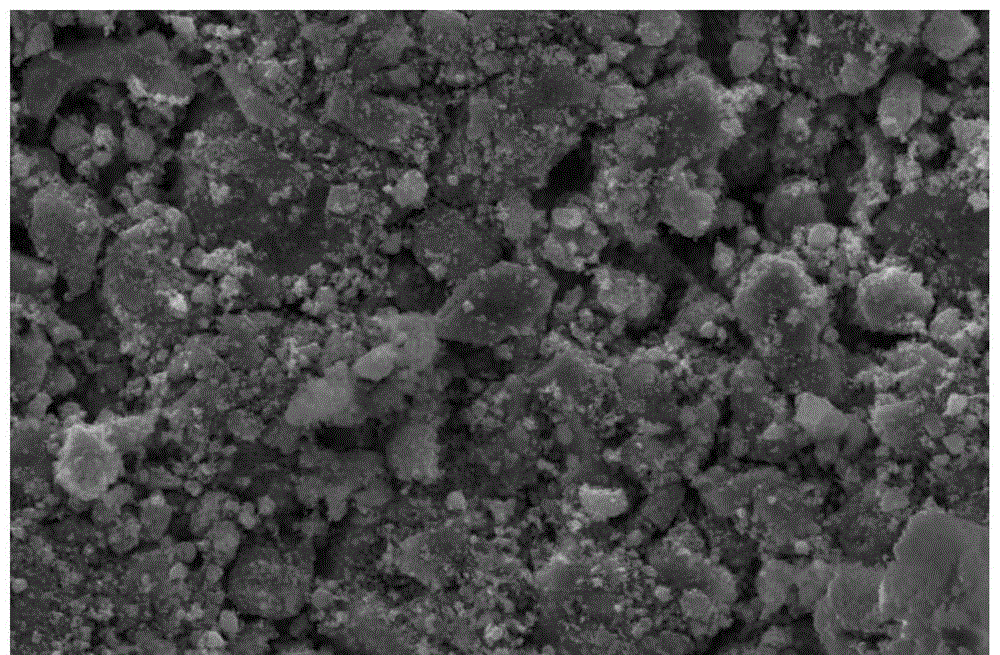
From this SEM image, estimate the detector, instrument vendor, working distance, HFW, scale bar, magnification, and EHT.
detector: SE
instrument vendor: FEI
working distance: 11.1 mm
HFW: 138 µm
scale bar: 50000 nm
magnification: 3 K X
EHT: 20 kV